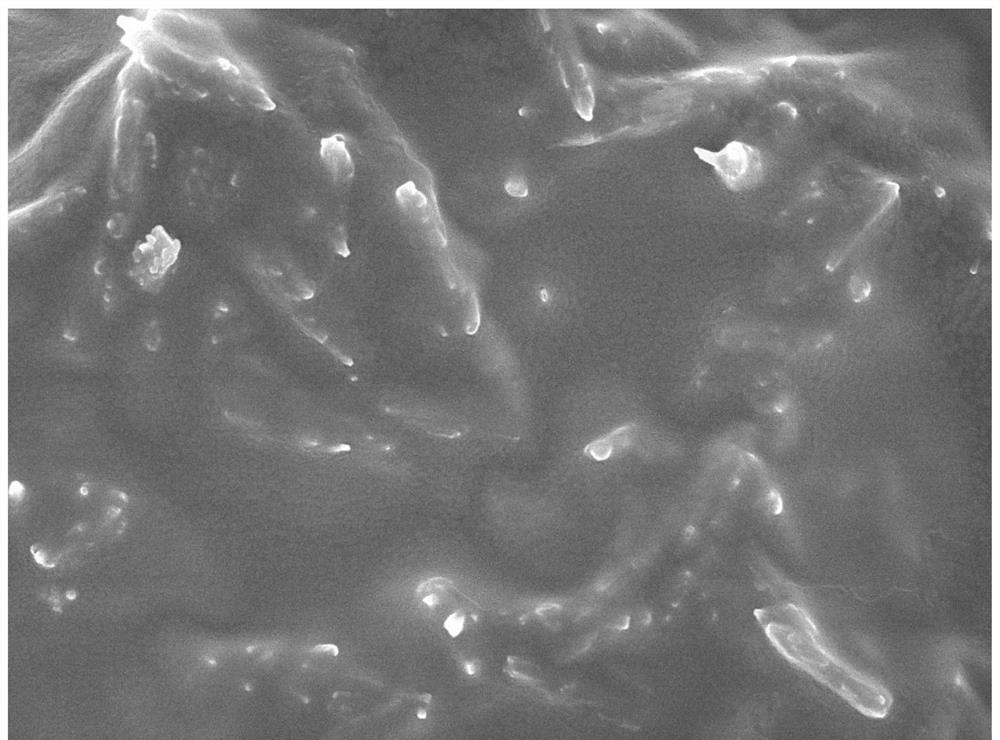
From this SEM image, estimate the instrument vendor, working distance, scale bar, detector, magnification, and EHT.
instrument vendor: JEOL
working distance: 8.1 mm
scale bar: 1000 nm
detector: SEI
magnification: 20 K X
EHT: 5 kV